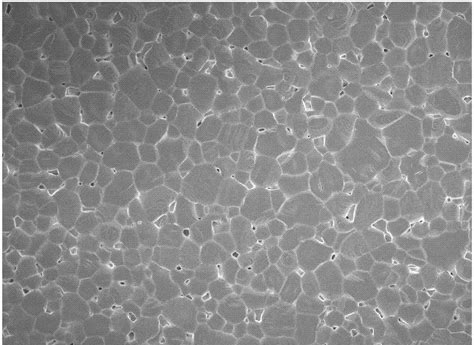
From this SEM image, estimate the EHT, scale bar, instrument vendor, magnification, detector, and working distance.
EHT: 5 kV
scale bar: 10000 nm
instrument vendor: JEOL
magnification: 0.95 K X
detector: SEI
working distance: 6.7 mm